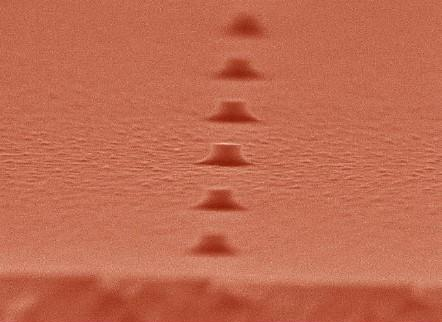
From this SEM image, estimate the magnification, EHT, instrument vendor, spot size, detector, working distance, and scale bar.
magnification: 80 K X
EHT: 20 kV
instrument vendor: FEI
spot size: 3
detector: TLD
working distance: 5 mm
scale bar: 200 nm